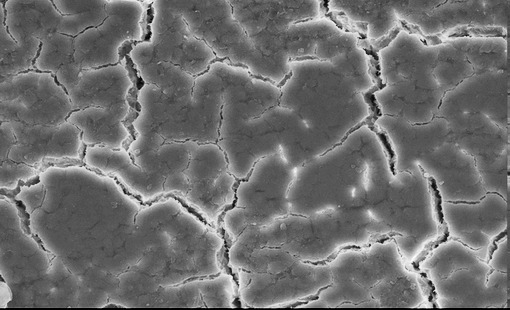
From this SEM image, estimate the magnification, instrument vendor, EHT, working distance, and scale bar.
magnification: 0.75 K X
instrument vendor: JEOL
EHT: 20 kV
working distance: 16 mm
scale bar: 10000 nm